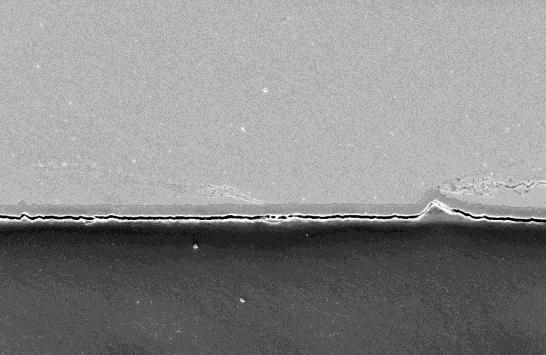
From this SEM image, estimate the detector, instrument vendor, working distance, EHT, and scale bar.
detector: InLens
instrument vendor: Zeiss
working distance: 9.2 mm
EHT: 10 kV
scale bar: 10000 nm